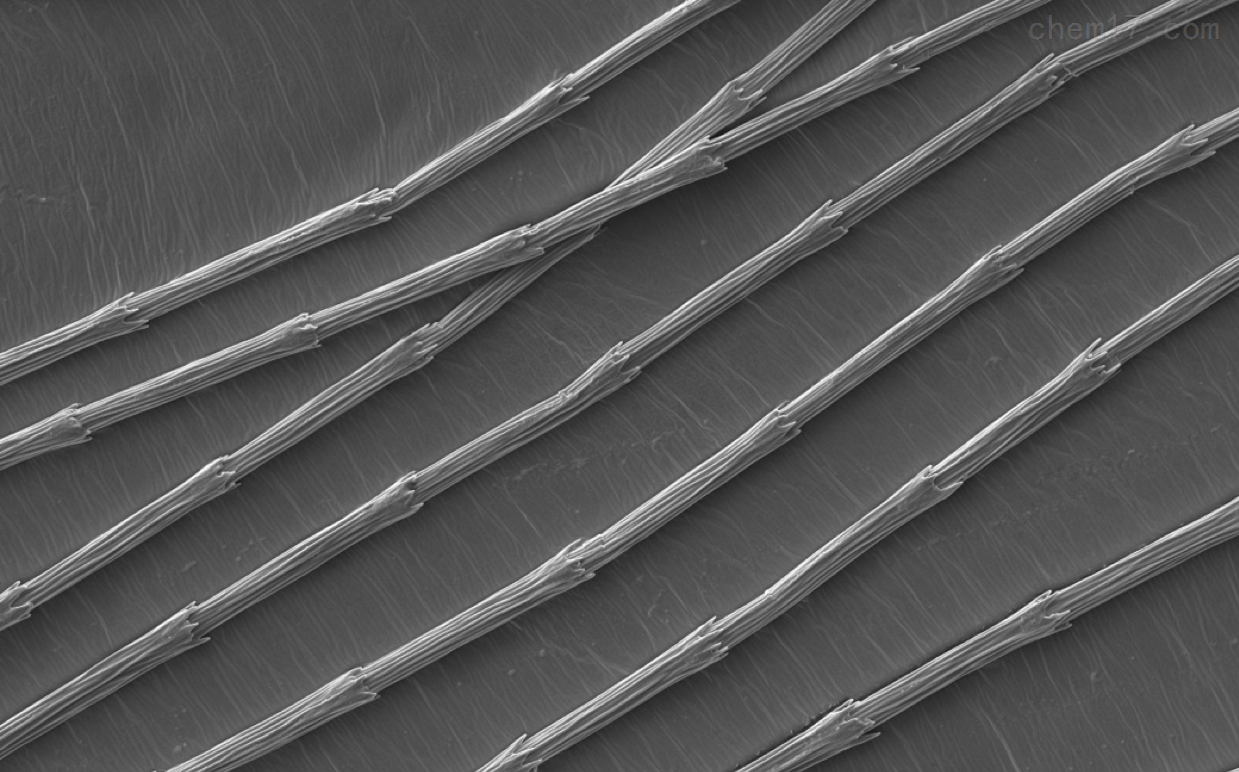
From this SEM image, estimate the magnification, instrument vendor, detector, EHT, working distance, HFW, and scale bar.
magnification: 0.5 K X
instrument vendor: Thermo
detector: SED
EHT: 5 kV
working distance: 5.943 mm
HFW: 254 µm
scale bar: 80000 nm